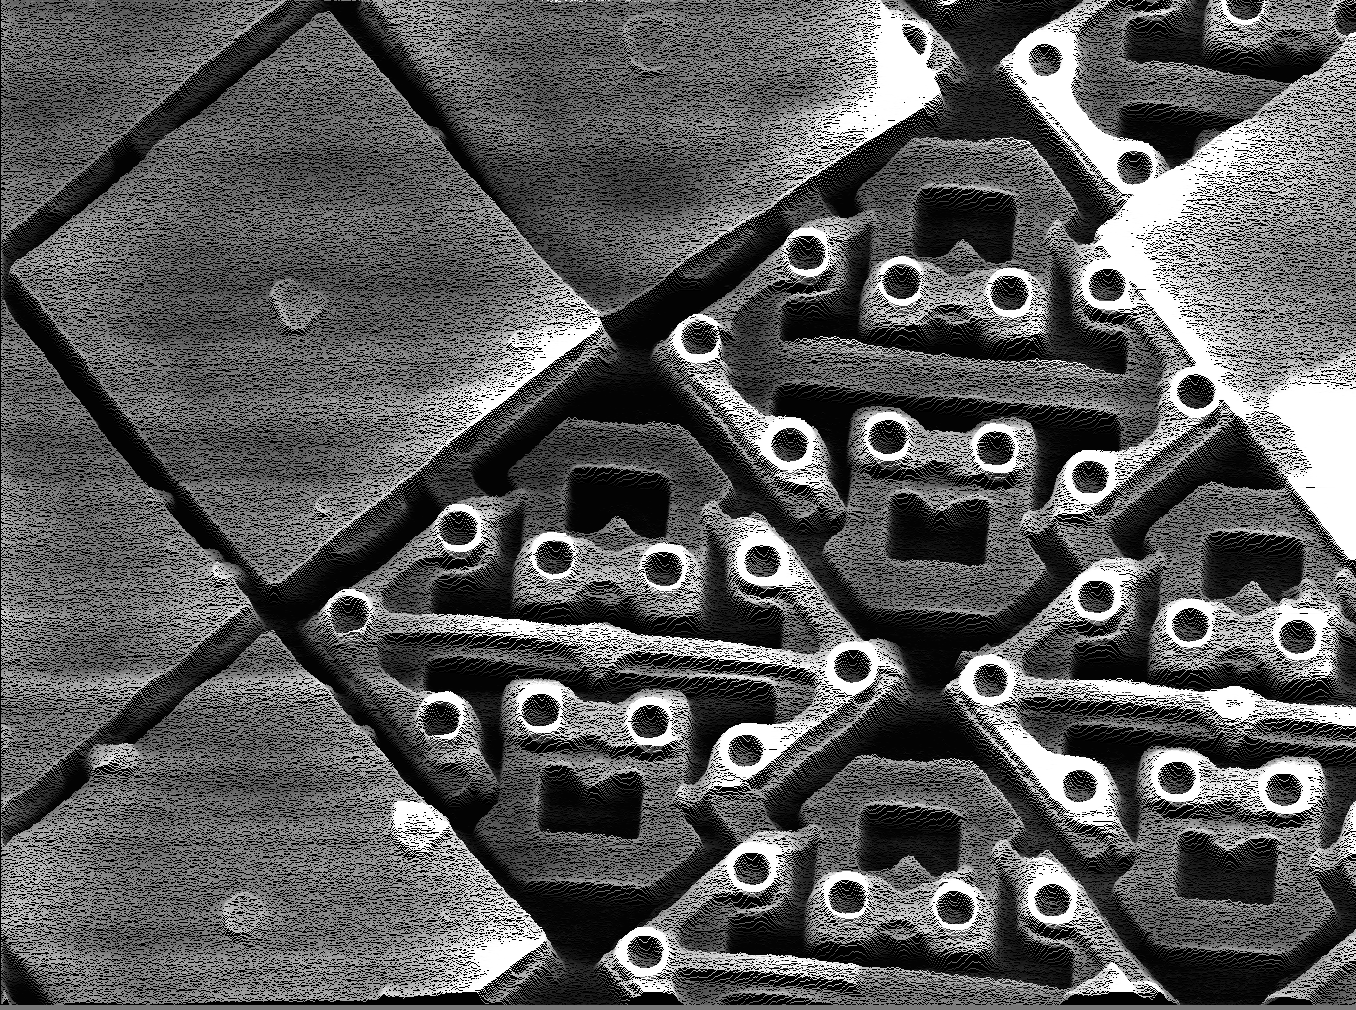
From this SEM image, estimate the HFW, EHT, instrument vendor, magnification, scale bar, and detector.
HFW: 40 µm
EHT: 15 kV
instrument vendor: other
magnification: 3 K X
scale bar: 3330 nm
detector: SE +Y-МОД